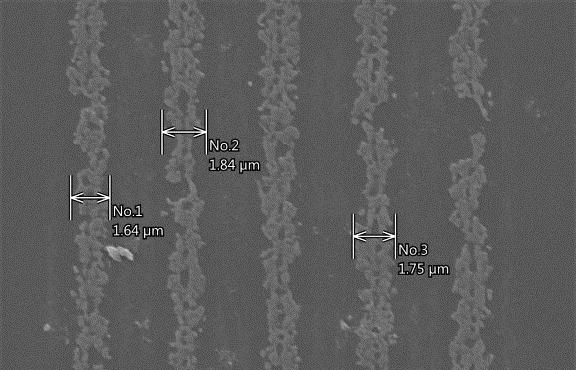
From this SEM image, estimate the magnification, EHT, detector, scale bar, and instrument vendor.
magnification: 5 K X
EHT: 15 kV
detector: SEI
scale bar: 5000 nm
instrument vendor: JEOL